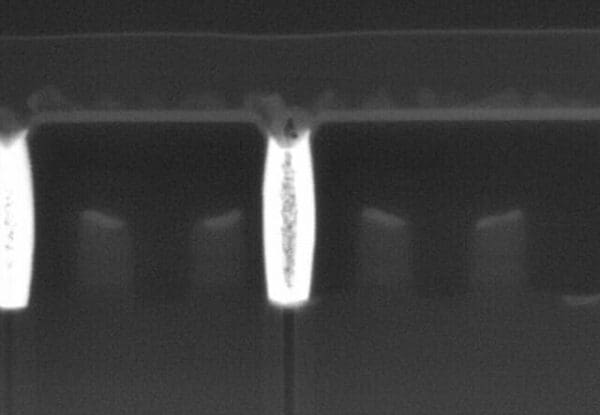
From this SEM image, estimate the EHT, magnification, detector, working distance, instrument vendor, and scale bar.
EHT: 10 kV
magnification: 45 K X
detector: YAGBSE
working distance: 10.1 mm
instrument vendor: Hitachi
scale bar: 1000 nm